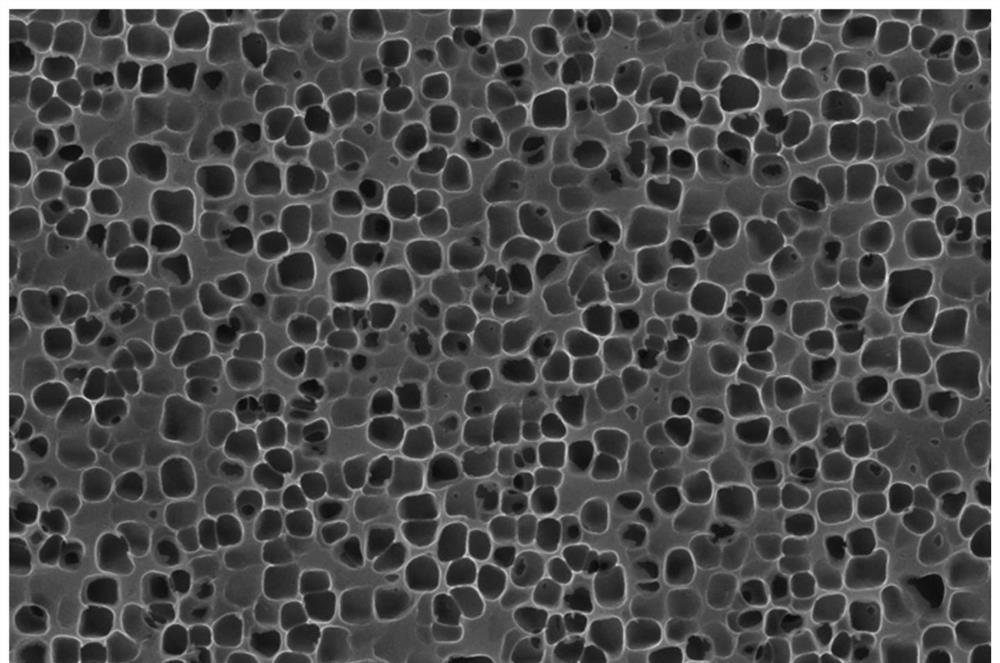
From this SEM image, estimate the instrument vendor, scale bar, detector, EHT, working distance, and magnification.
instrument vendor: Zeiss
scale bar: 200 nm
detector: InLens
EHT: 15 kV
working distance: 5.6 mm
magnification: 20 K X